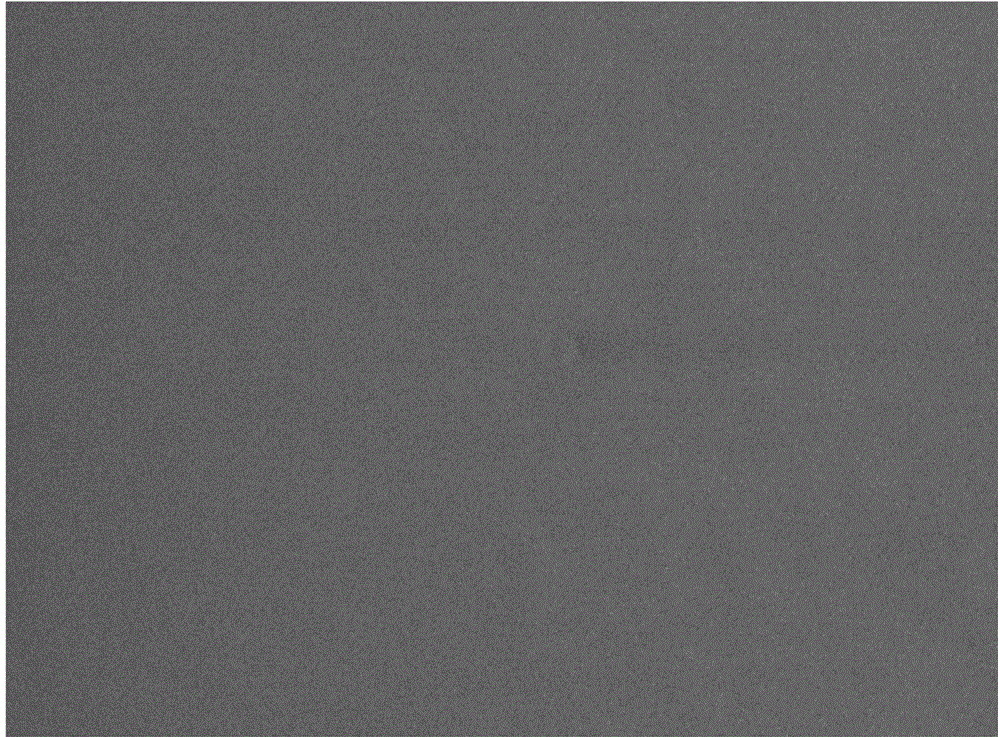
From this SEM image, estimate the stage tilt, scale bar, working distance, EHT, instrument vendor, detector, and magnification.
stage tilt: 0°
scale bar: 400 nm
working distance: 4.4 mm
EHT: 0.5 kV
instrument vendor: FEI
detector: TLD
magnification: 100 K X